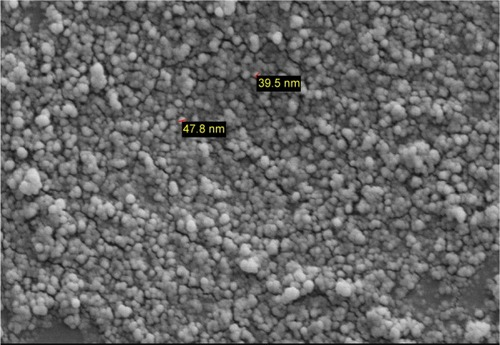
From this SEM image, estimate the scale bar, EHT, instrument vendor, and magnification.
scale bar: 1000 nm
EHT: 26 kV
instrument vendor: other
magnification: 40 K X